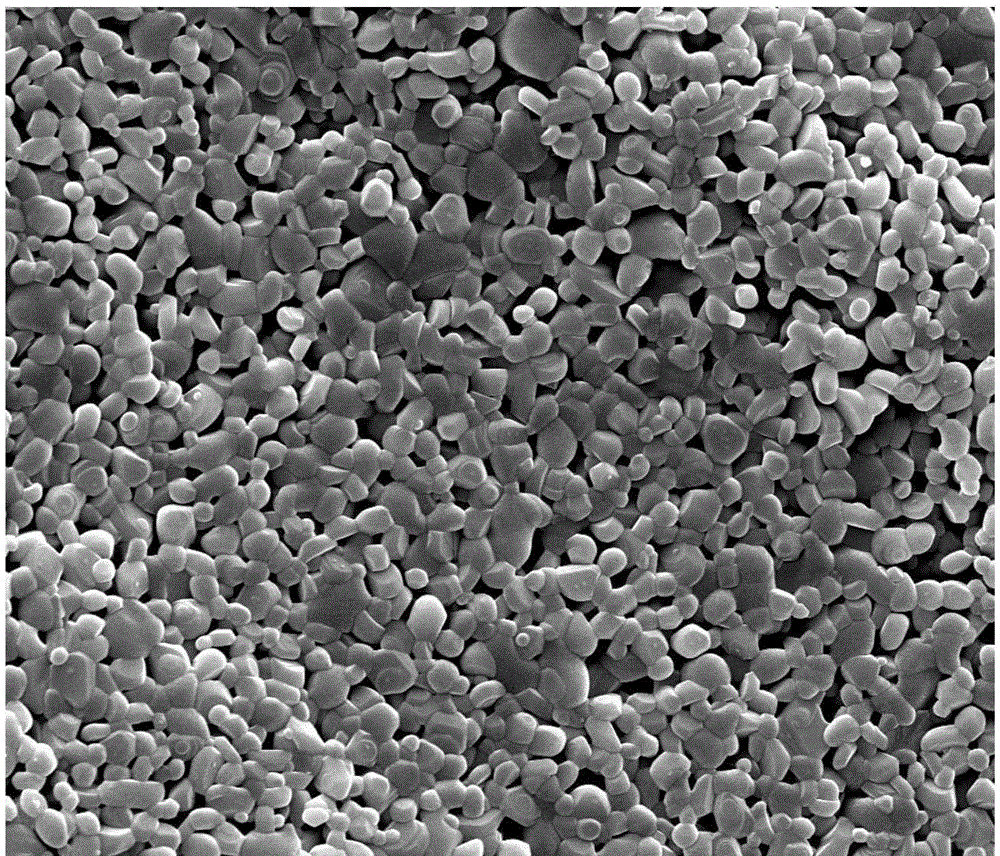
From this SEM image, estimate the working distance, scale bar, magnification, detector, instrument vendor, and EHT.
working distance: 8.9 mm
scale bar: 10000 nm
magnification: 10 K X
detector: SE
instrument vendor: FEI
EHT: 20 kV